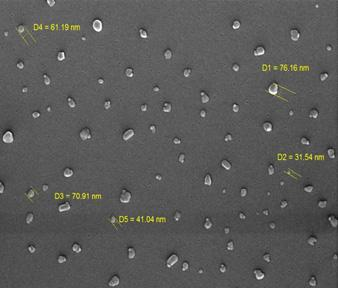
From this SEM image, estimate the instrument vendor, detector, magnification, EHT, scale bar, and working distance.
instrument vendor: Tescan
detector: InBeam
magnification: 100 K X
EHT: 20 kV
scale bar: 500 nm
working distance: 4.574 mm